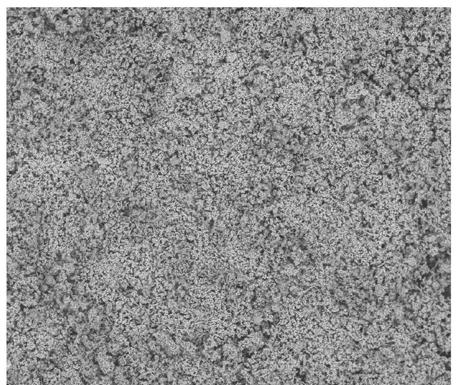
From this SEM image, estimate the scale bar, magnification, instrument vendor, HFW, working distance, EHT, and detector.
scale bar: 100000 nm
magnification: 0.5 K X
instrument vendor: FEI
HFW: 600 µm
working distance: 5.5 mm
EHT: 15 kV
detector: BSED